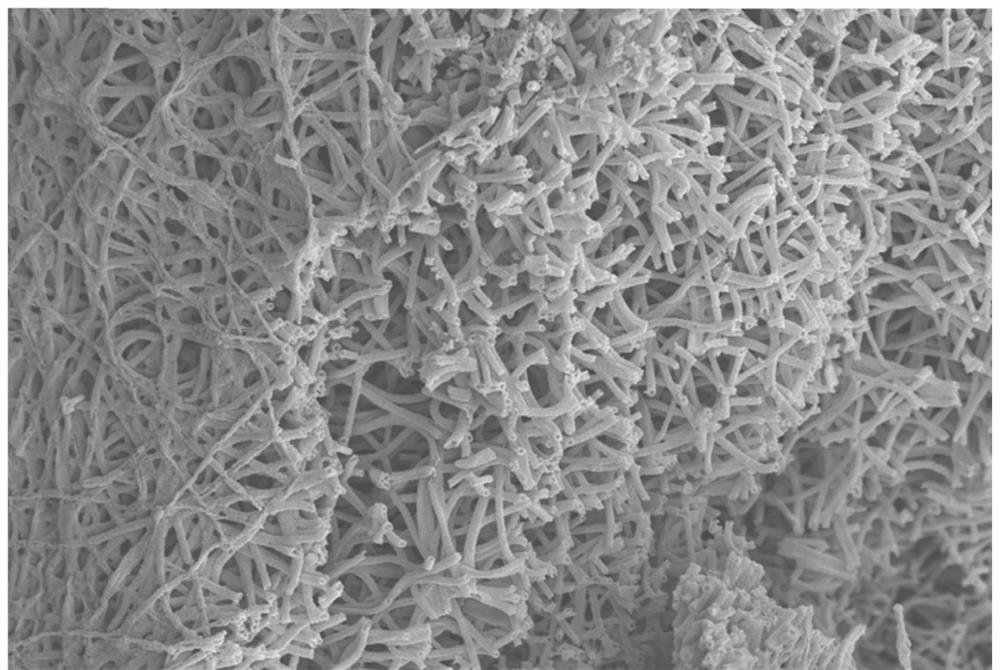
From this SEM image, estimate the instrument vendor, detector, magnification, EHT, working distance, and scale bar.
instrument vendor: Zeiss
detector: InLens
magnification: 5 K X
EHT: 5 kV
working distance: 7.9 mm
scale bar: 1000 nm